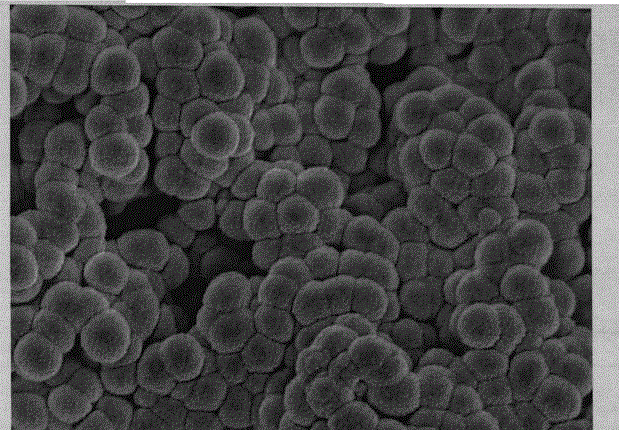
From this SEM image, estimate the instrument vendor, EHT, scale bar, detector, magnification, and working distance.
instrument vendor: JEOL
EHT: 5 kV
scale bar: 100 nm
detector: SEI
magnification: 30 K X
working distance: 9 mm